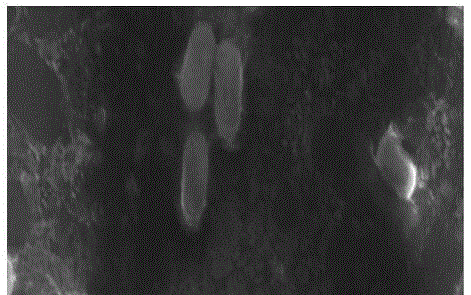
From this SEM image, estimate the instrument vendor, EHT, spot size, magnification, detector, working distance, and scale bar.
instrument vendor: FEI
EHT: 20 kV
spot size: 3.5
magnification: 12 K X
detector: ETD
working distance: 9.8 mm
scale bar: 5000 nm